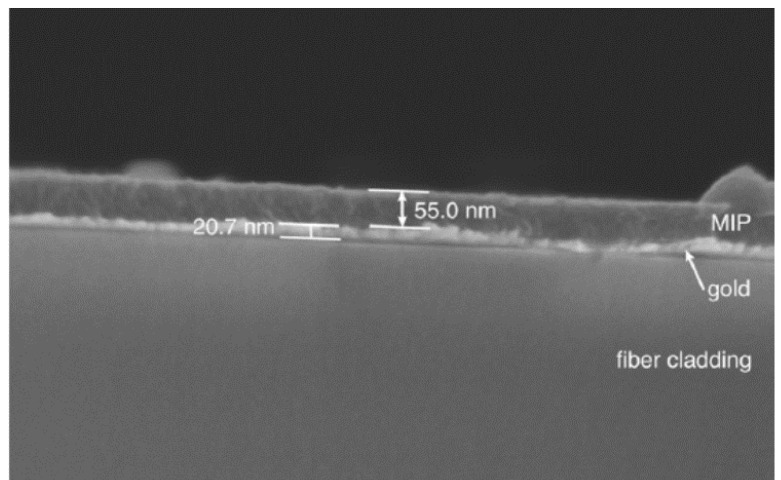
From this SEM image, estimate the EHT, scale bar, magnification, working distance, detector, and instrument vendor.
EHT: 3 kV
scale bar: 500 nm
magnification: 110 K X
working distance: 2.4 mm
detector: SE(U)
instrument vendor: Hitachi